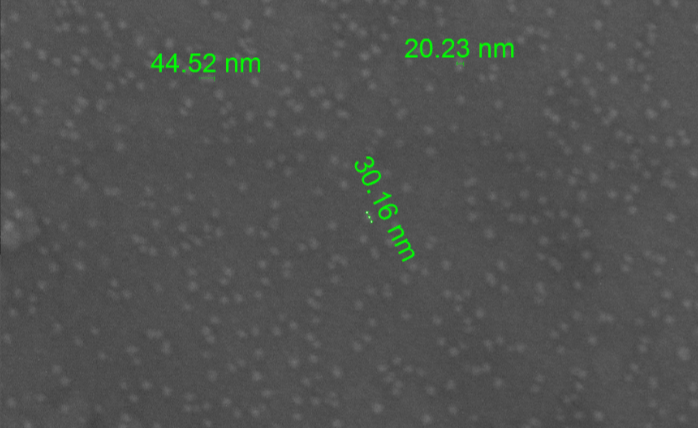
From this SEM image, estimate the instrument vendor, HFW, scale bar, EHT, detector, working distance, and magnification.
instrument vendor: FEI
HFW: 2.07 µm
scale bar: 500 nm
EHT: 20 kV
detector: SE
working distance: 10.4 mm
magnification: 200 K X